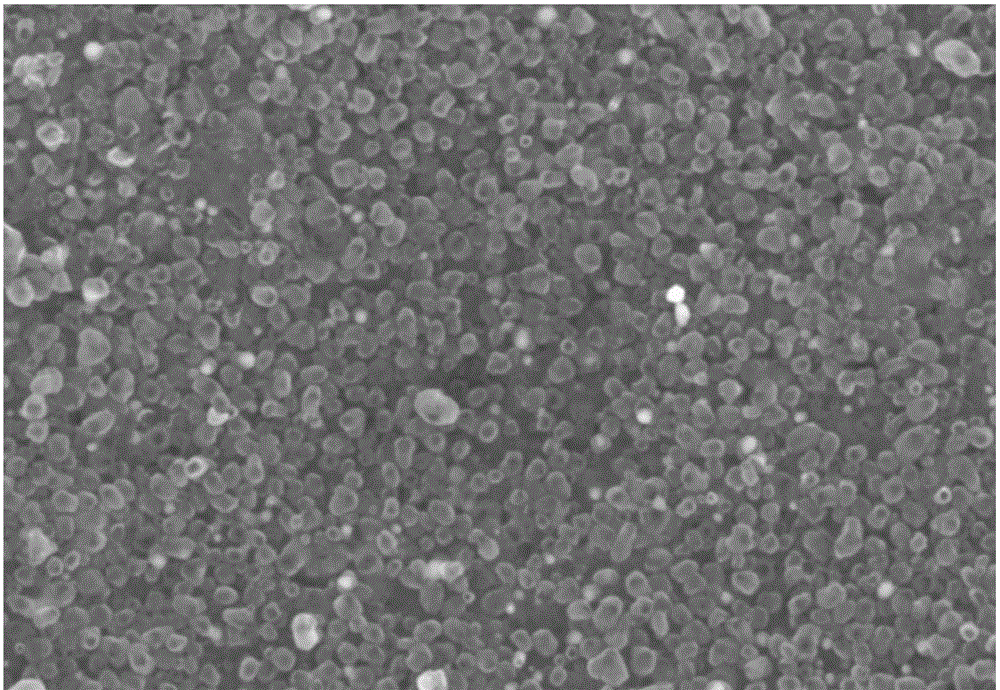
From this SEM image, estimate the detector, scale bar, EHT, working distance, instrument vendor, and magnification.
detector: SE(UL)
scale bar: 500 nm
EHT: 5 kV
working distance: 10 mm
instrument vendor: Hitachi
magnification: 60 K X